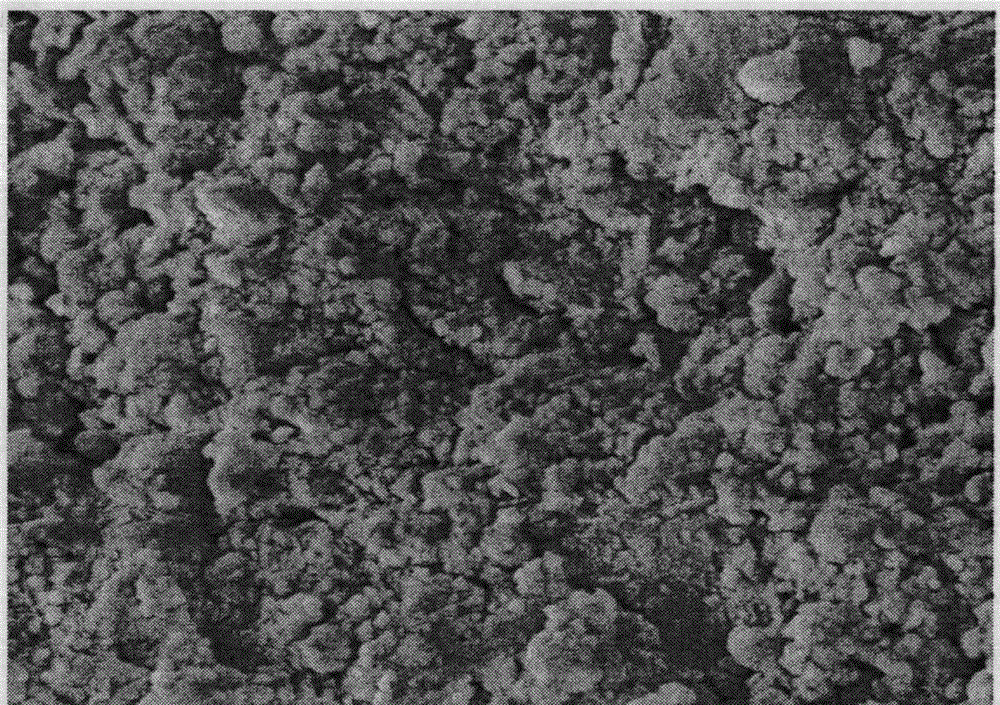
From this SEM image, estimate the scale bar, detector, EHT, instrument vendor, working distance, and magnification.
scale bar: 1000 nm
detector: SEI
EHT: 5 kV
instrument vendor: JEOL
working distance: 5 mm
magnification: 20 K X